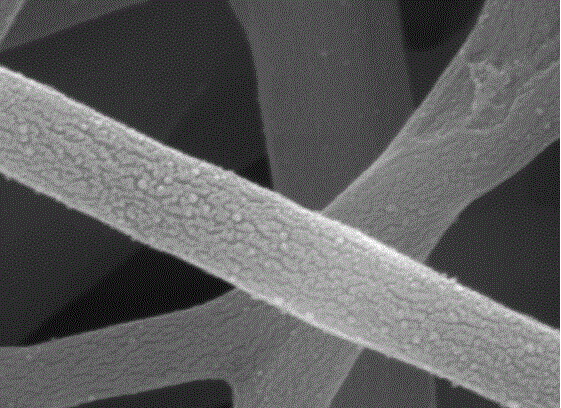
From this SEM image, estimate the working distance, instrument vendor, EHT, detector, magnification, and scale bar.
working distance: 8.8 mm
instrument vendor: JEOL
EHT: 10 kV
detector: SEI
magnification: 100 K X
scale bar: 100 nm